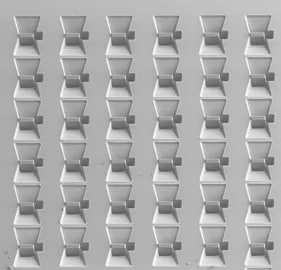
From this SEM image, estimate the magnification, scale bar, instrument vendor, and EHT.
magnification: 0.37 K X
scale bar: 60000 nm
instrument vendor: JEOL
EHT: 2 kV